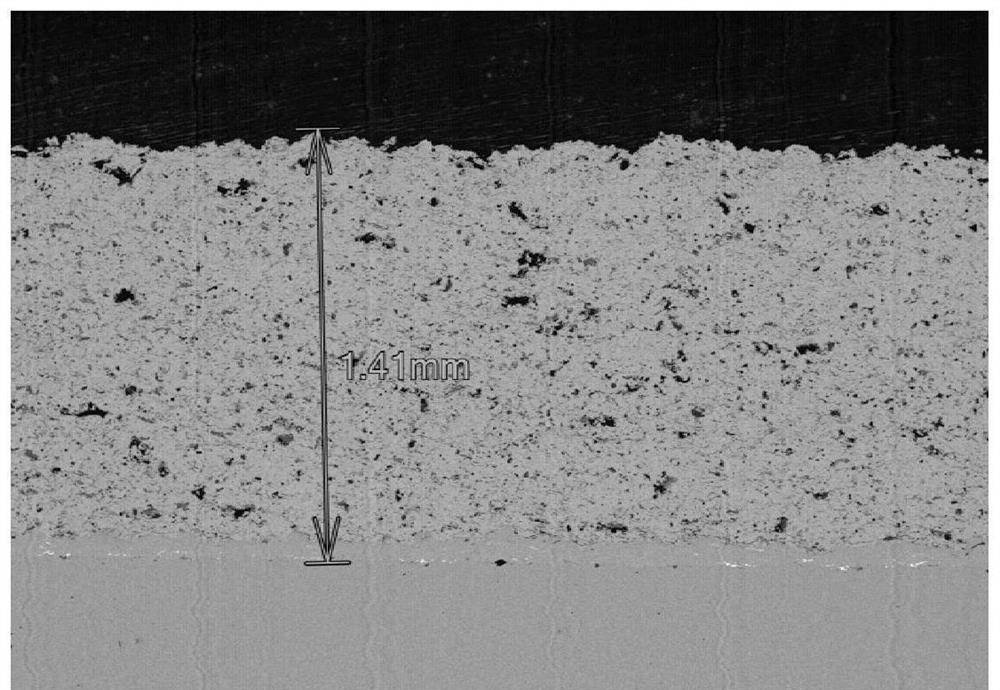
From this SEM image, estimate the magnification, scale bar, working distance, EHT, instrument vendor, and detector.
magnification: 0.04 K X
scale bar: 1000000 nm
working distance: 24.6 mm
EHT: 15 kV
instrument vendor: Hitachi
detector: BSE-ALL